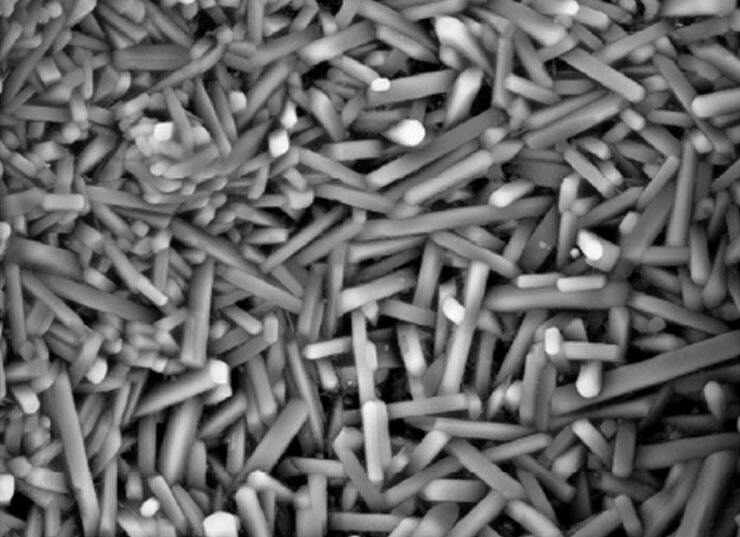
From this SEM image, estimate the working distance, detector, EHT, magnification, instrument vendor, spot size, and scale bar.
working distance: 6 mm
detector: BSED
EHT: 20 kV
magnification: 5 K X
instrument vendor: FEI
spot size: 5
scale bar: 10000 nm